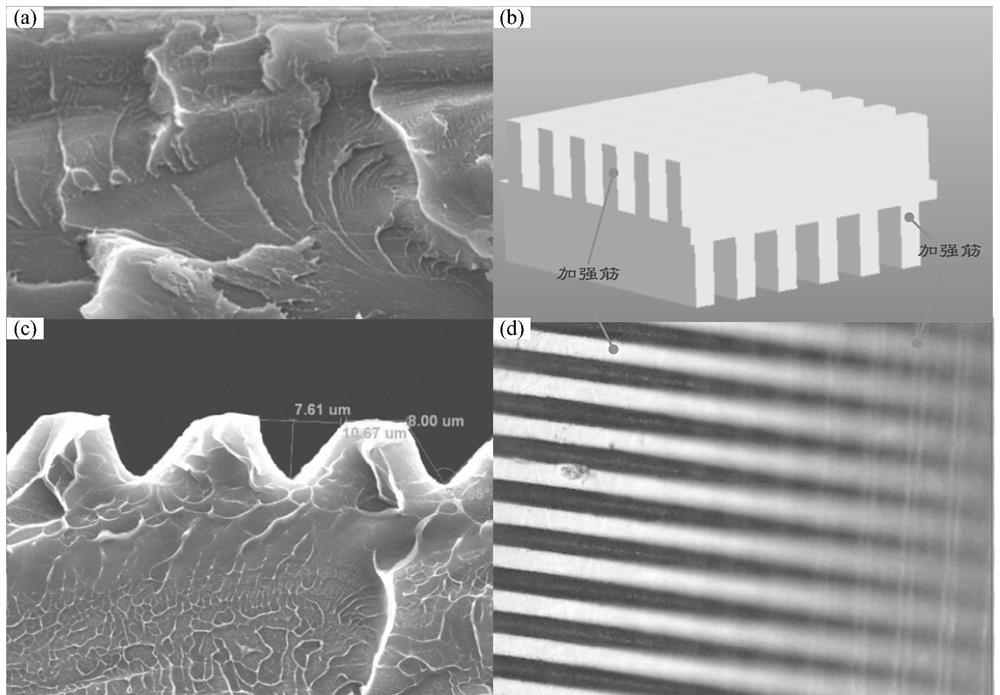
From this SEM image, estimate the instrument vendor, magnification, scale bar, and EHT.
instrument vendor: JEOL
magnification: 1.5 K X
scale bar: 10000 nm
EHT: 20 kV